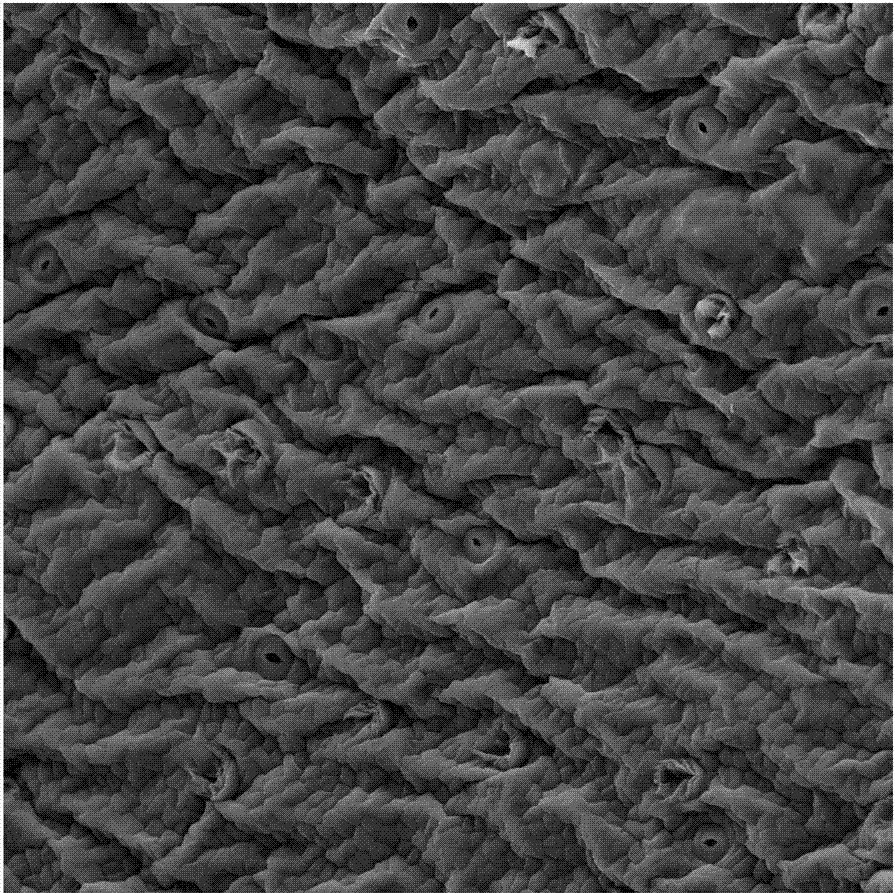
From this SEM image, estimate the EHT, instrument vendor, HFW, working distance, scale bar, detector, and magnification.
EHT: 30 kV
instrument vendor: Tescan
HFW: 433 µm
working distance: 17.16 mm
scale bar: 100000 nm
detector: SE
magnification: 0.5 K X